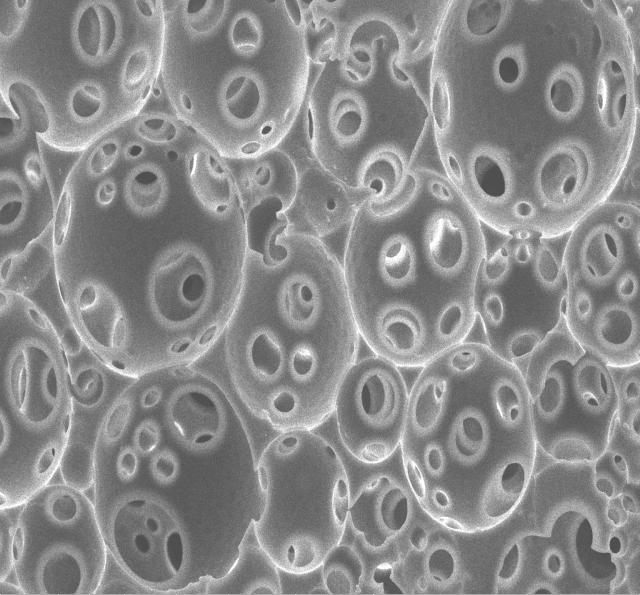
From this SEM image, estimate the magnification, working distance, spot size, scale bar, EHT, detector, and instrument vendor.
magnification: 2.5 K X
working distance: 9.4 mm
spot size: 3.5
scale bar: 50000 nm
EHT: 10 kV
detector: ETD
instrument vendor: FEI